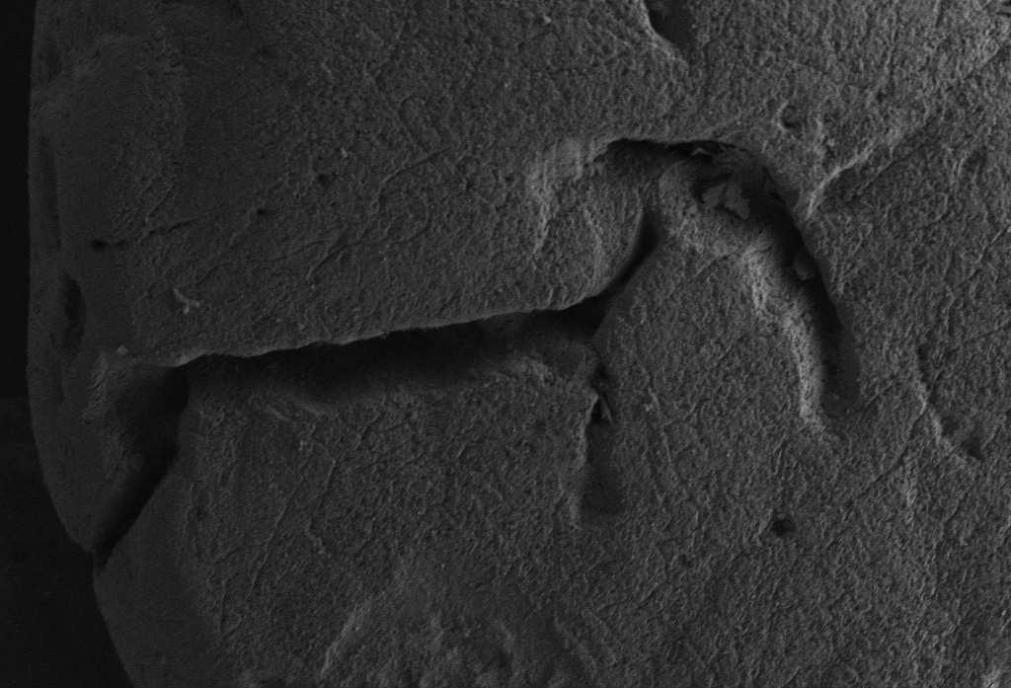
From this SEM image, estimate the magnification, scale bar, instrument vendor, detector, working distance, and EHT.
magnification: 1 K X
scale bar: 20000 nm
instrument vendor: Zeiss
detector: SE2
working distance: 5.1 mm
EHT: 3 kV